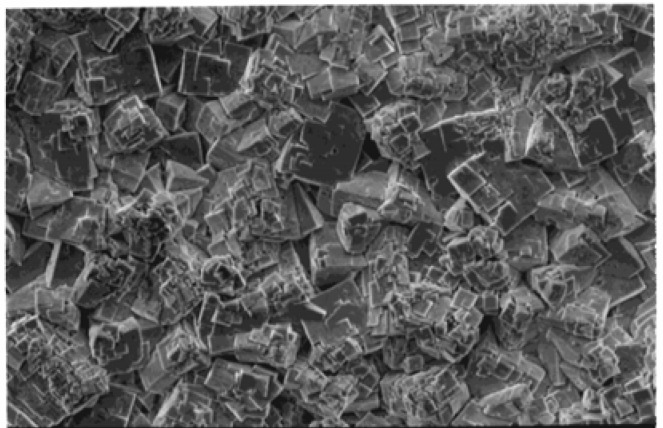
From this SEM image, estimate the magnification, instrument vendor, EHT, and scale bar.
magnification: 2 K X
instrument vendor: JEOL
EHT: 10 kV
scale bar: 10000 nm